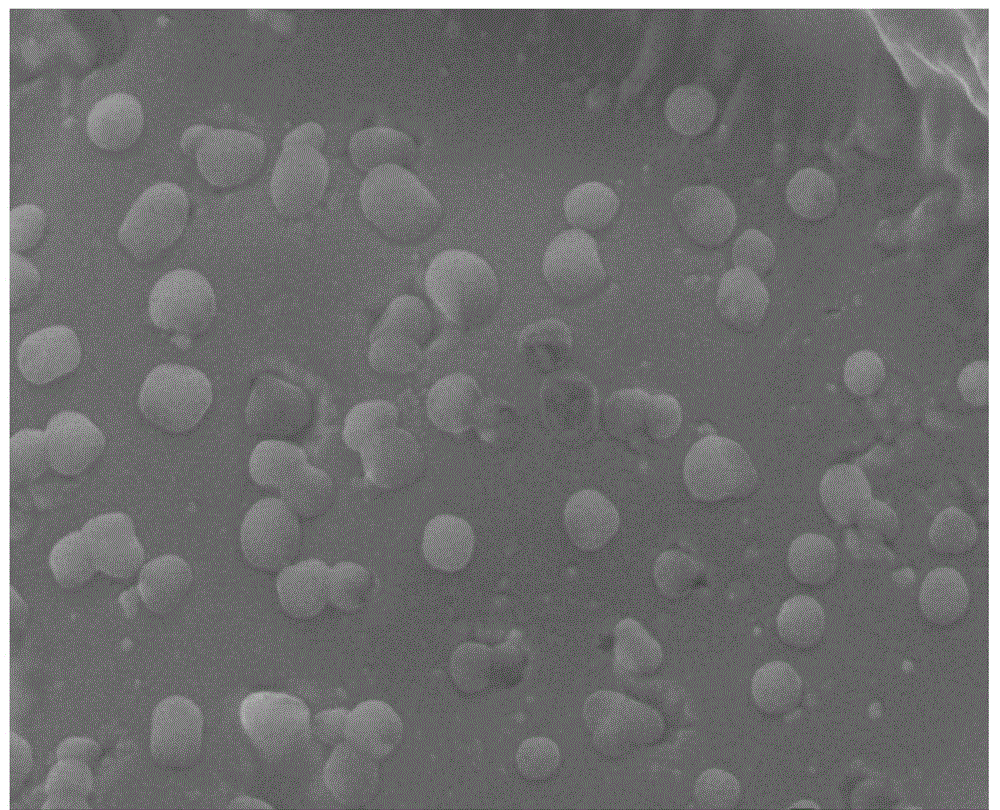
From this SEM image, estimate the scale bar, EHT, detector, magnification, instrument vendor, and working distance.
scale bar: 100 nm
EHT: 10 kV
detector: SEI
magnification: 20 K X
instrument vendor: JEOL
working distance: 18 mm